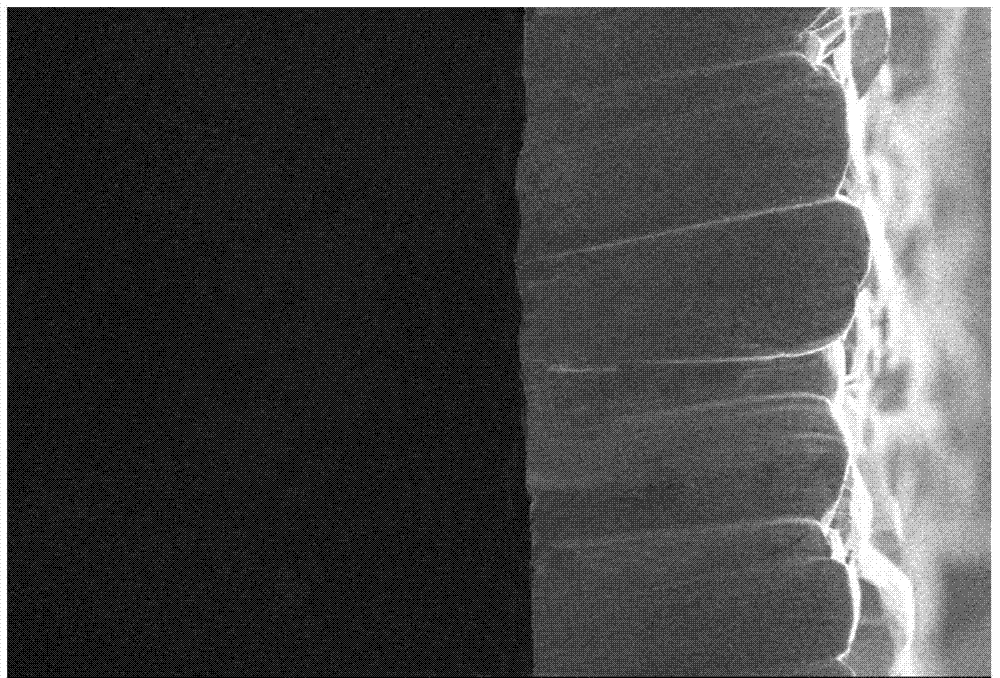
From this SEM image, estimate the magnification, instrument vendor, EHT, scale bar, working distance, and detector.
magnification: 0.3 K X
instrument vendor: JEOL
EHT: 10 kV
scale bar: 50000 nm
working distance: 11 mm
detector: SEI